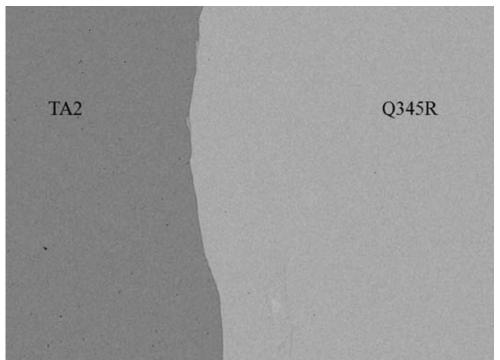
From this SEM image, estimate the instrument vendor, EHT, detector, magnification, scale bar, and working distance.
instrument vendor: JEOL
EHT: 20 kV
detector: BED-C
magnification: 0.2 K X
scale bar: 100000 nm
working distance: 9.9 mm